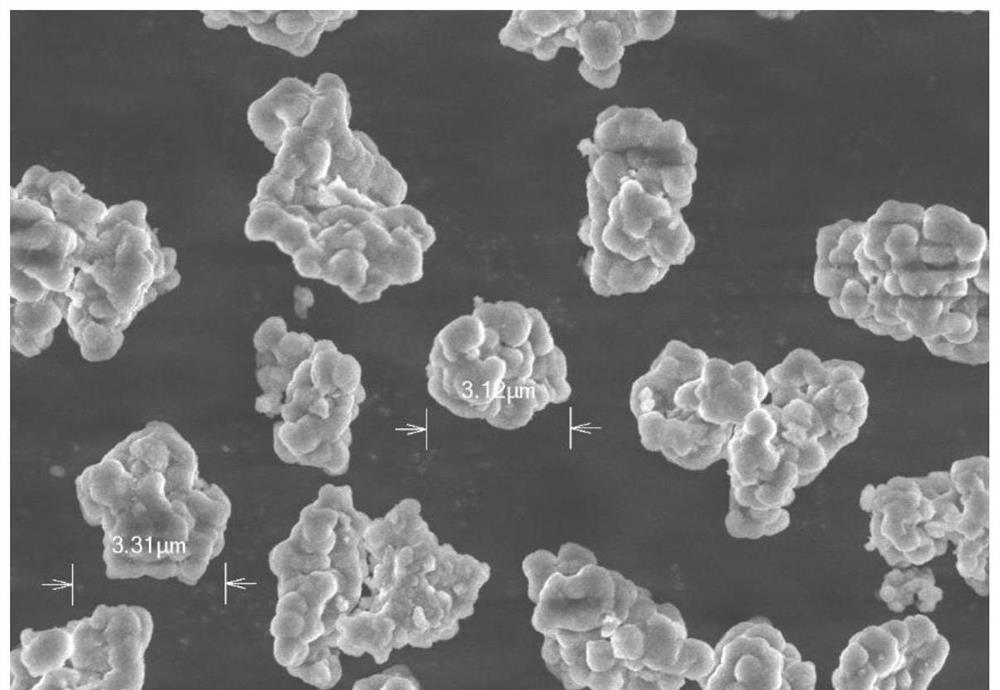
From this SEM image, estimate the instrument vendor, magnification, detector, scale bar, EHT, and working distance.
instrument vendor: Hitachi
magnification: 6 K X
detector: SE(UL)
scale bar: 5000 nm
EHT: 5 kV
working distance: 6.9 mm